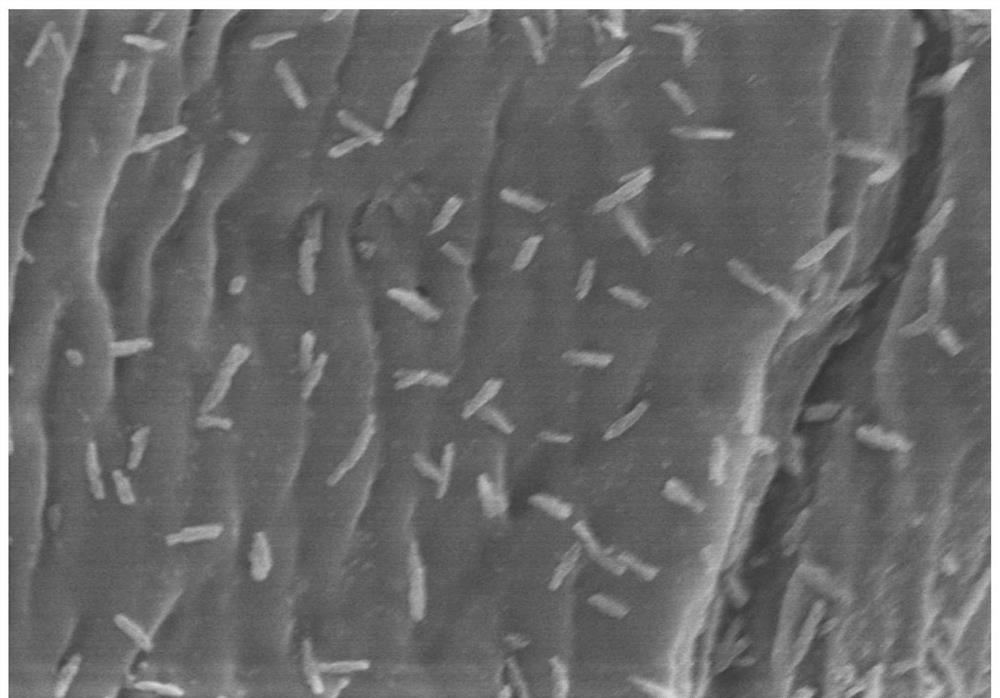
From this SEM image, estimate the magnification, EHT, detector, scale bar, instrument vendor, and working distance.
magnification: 20 K X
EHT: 5 kV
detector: SE(M)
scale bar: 2000 nm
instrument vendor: Hitachi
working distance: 7 mm